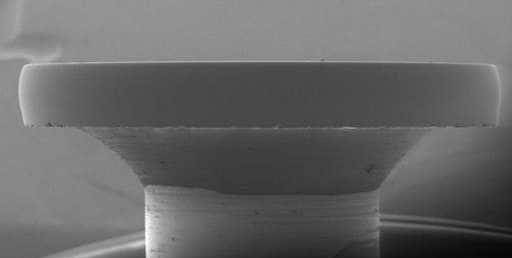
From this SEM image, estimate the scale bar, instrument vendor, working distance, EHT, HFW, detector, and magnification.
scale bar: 2000000 nm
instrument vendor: Thermo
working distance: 13.4 mm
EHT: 2 kV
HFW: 4140 µm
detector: LVD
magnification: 0.1 K X